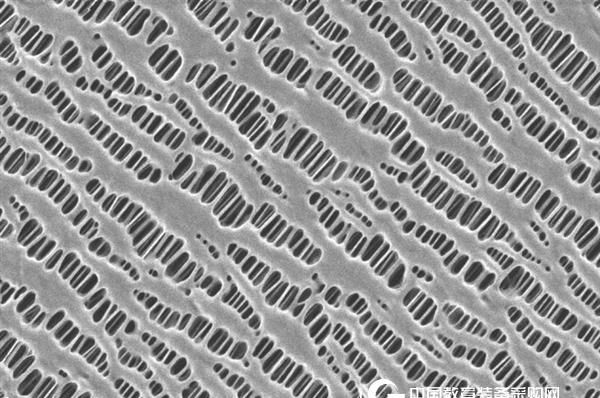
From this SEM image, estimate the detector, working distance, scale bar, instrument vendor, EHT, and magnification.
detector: InLens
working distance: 1.2 mm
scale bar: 200 nm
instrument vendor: Zeiss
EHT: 0.5 kV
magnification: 20 K X